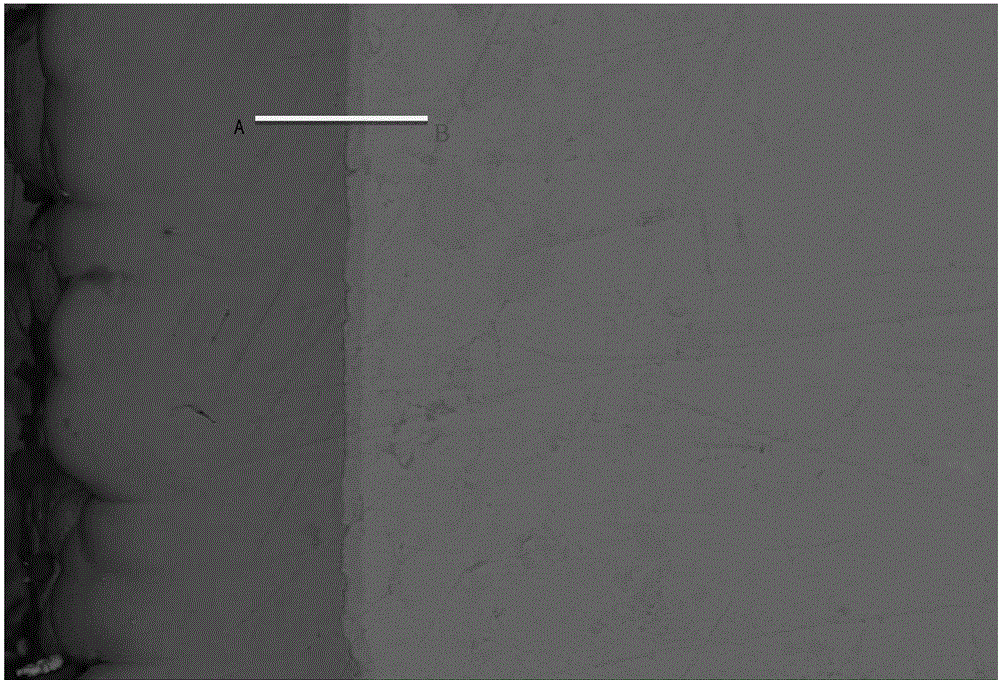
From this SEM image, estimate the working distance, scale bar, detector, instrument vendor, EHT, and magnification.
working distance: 7 mm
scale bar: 10000 nm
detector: AsB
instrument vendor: Zeiss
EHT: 15 kV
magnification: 1 K X